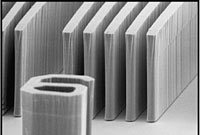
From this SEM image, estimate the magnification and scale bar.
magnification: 1.4 K X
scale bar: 10000 nm